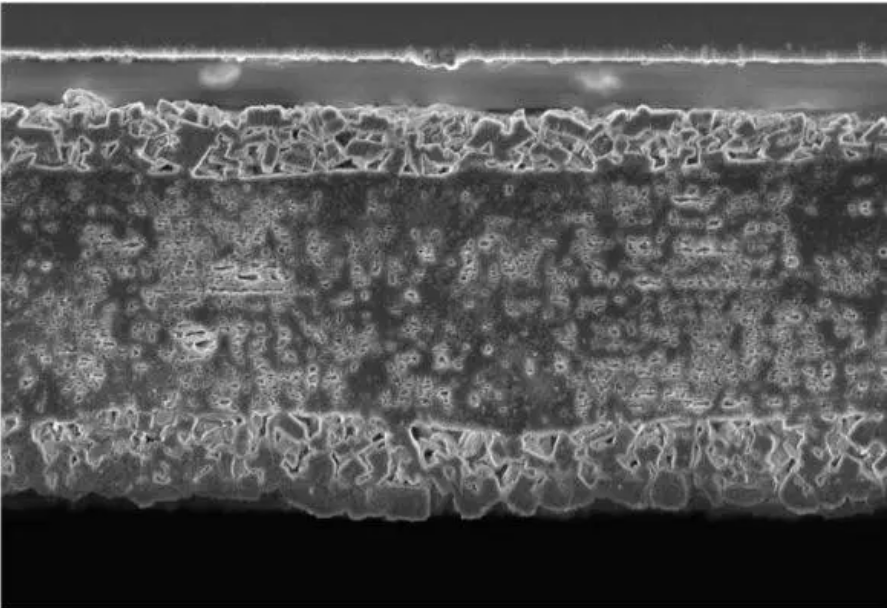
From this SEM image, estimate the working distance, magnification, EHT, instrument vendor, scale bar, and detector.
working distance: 2.3 mm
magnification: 5 K X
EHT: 3 kV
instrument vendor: Zeiss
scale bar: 30000 nm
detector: InLens